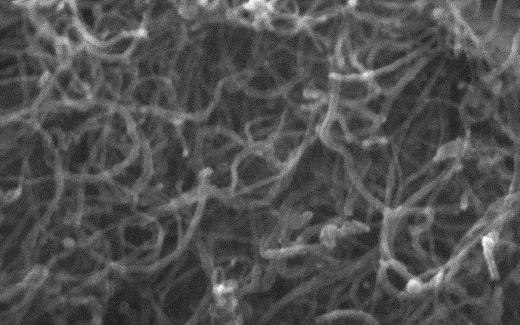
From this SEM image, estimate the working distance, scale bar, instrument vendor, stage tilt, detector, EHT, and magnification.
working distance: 6.8 mm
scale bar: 200 nm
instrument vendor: Zeiss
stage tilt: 0°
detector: InLens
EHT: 15 kV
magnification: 200 K X